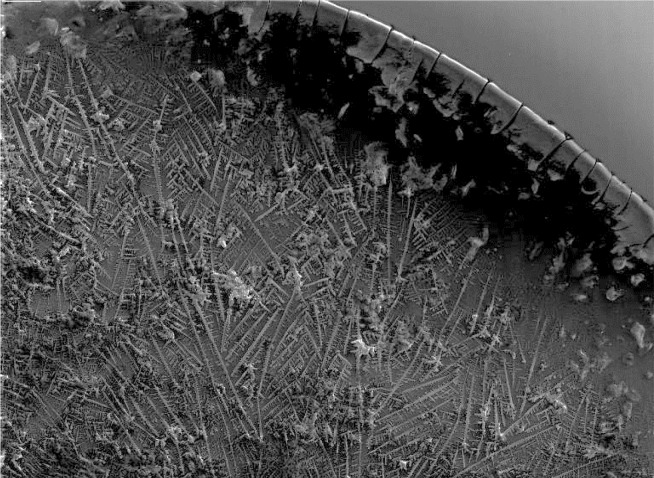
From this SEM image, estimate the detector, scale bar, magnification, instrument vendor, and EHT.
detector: SE Detector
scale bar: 1000000 nm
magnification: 0.07 K X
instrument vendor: Tescan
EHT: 20 kV